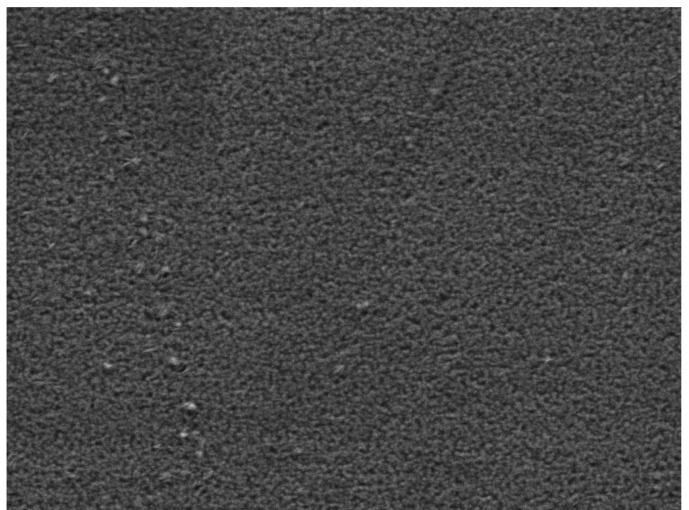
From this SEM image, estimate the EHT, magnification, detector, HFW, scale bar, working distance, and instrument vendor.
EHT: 5 kV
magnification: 60 K X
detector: SE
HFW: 4.61 µm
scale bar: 1000 nm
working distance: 2.9 mm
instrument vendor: Tescan